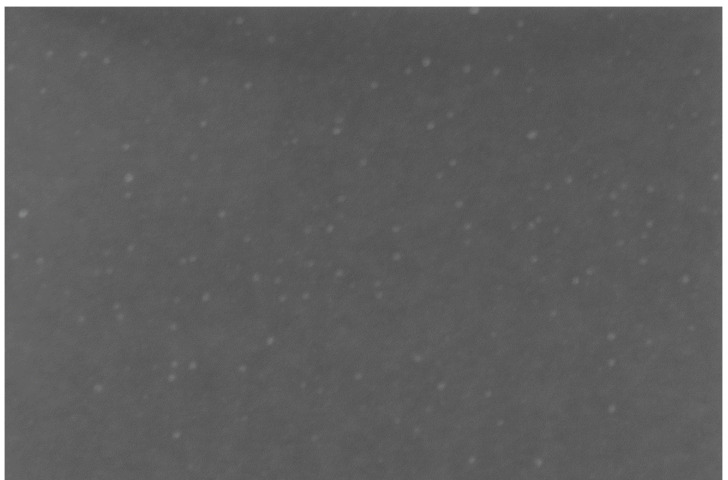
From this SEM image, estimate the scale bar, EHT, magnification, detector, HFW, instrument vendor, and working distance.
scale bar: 500 nm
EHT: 2 kV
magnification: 80.035 K X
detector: TLD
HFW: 1.86 µm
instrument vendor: FEI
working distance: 2.7 mm